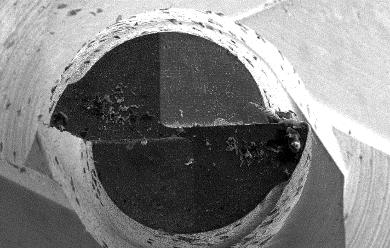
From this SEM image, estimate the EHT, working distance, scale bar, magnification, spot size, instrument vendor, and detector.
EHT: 15 kV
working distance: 14.3 mm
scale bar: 200000 nm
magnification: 0.15 K X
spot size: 3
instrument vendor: FEI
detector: SE